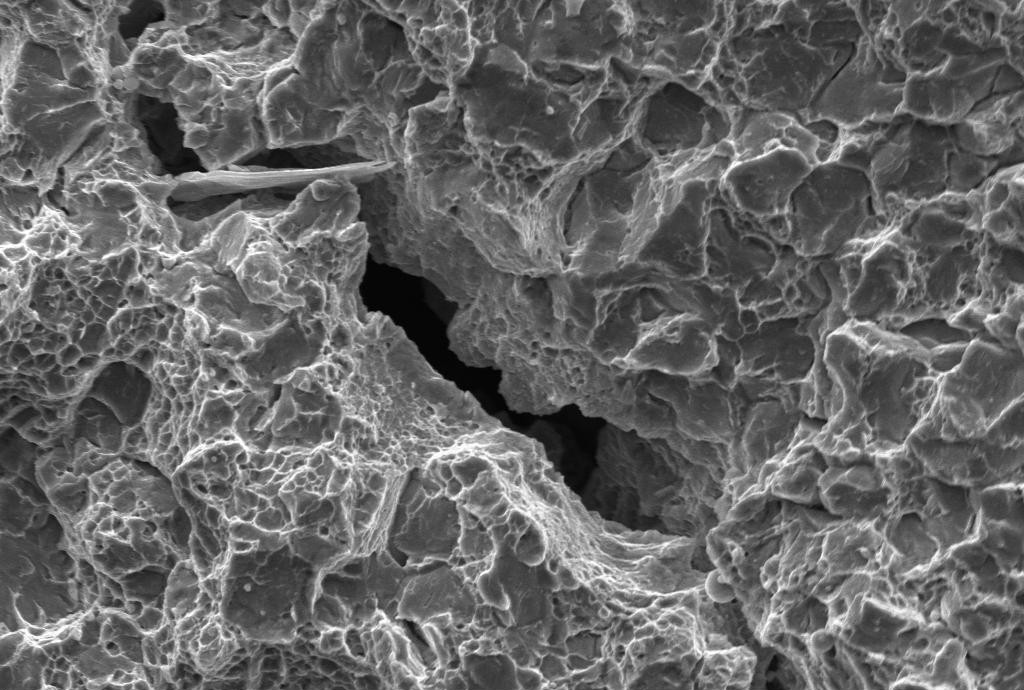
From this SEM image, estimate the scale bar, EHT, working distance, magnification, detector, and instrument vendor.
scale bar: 10000 nm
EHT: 20 kV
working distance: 14.5 mm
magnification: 1 K X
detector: SE1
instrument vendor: Zeiss